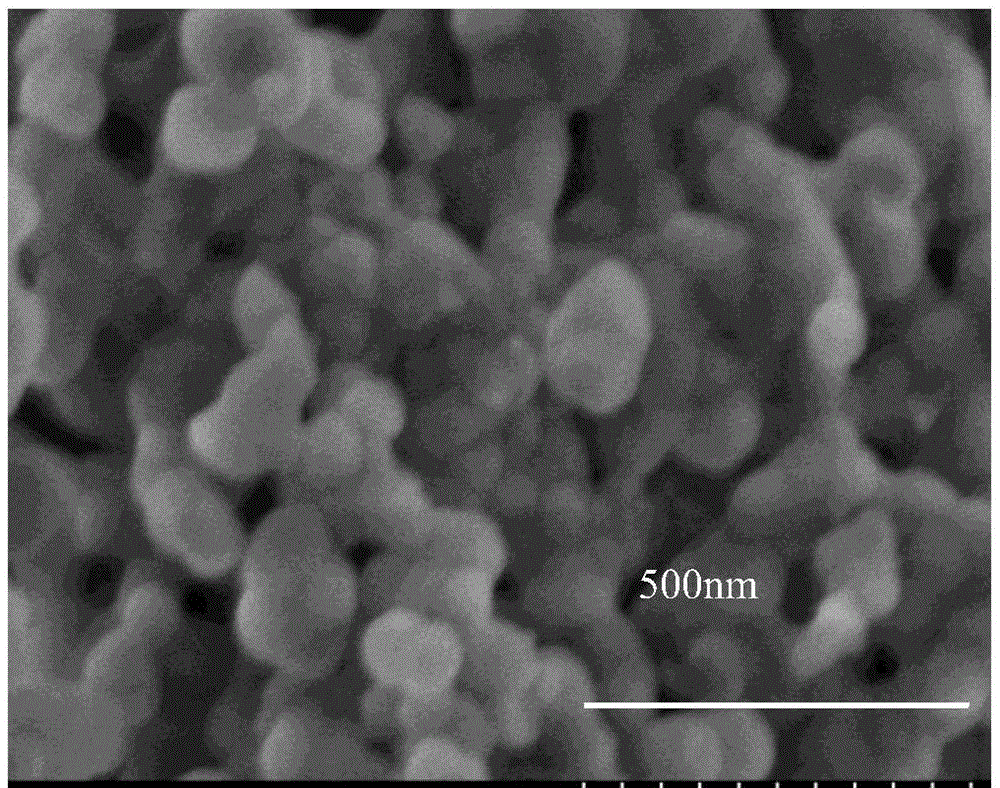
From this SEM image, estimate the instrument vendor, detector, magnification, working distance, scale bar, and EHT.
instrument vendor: Hitachi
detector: SE(U)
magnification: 100 K X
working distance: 7.9 mm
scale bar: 500 nm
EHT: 3 kV